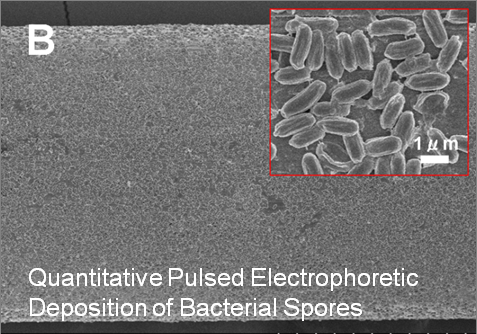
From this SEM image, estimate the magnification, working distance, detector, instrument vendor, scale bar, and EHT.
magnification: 1 K X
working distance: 13.5 mm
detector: SE(U)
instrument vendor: Hitachi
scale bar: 50000 nm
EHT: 5 kV